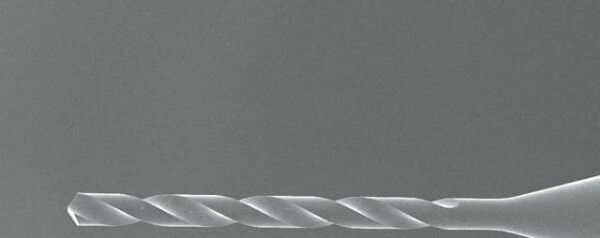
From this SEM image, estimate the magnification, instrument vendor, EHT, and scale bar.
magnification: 0.15 K X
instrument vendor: JEOL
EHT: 15 kV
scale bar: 100000 nm